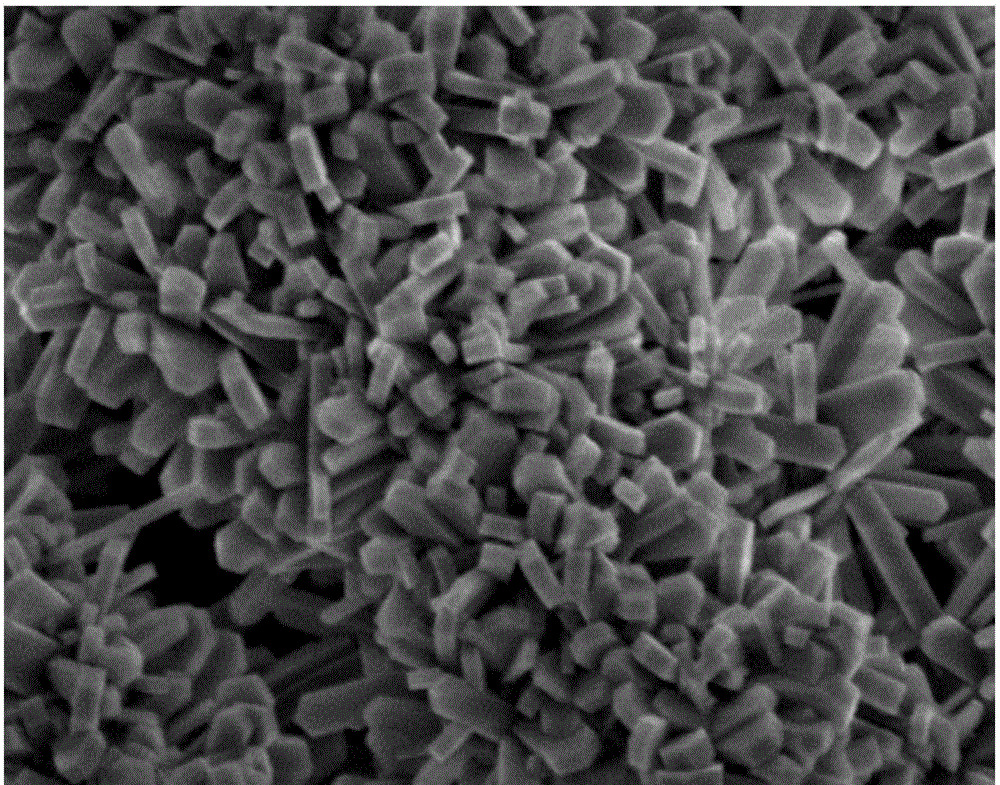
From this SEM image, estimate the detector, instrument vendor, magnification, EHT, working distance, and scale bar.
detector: SEI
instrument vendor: JEOL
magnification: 20 K X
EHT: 3 kV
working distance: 9.3 mm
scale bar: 1000 nm